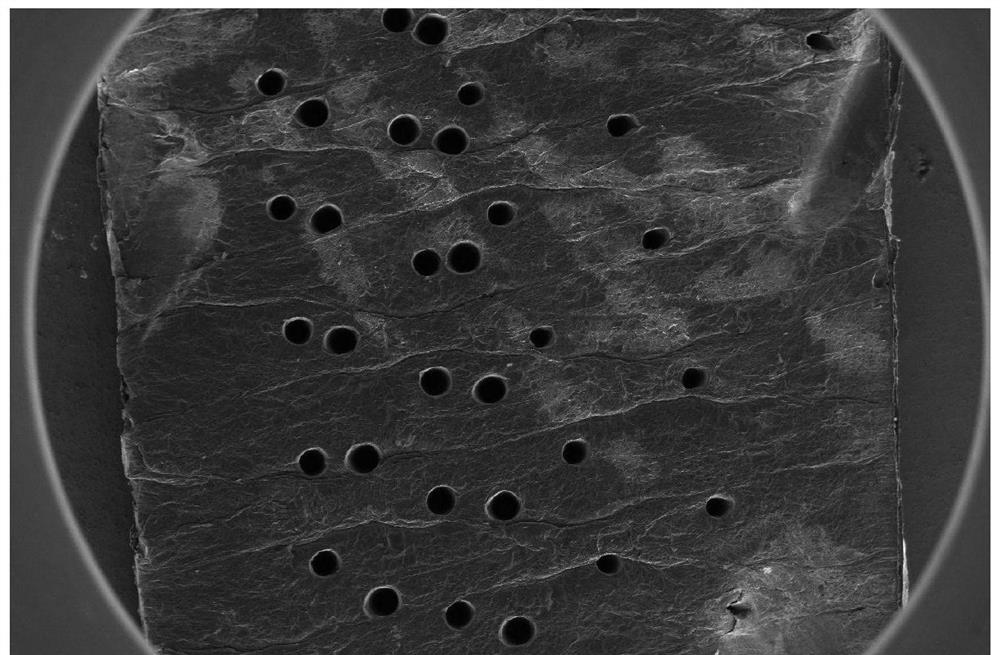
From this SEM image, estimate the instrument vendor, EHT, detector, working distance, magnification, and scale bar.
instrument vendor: FEI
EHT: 5 kV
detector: ETD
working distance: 5.5 mm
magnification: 0.051 K X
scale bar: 1000000 nm